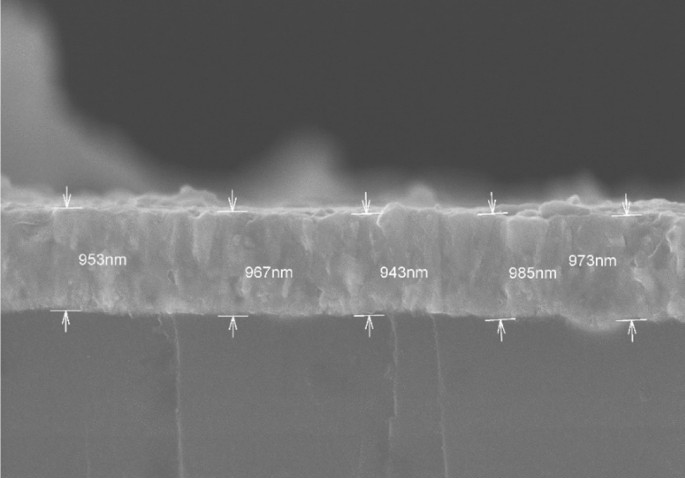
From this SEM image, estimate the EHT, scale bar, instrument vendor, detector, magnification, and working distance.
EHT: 10 kV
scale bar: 2000 nm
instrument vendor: Hitachi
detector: SE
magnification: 20 K X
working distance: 10.6 mm